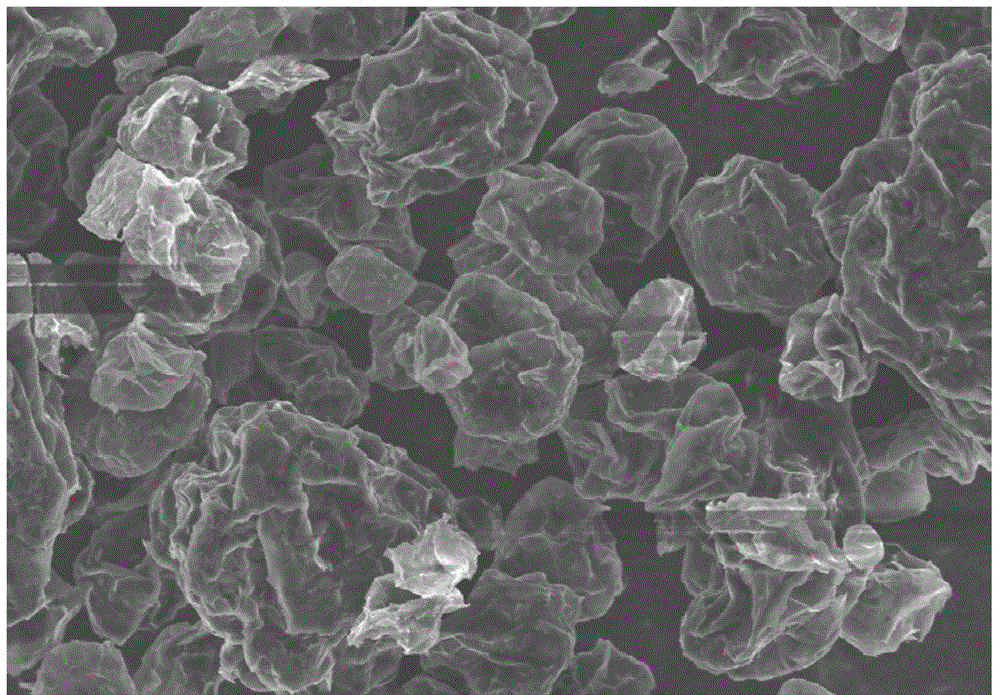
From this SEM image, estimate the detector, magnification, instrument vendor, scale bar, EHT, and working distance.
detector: SE(M)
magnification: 1 K X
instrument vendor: Hitachi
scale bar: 50000 nm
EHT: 8 kV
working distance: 9.2 mm